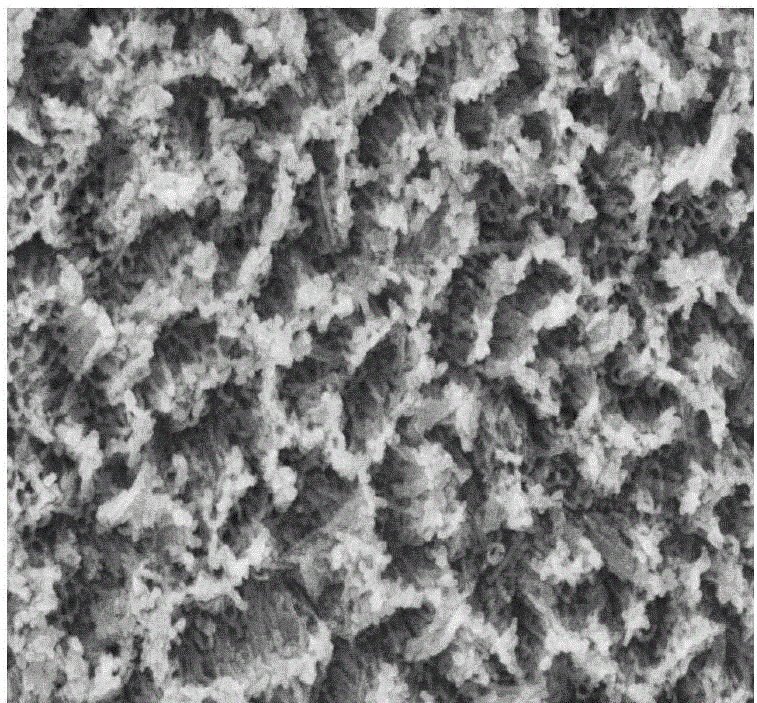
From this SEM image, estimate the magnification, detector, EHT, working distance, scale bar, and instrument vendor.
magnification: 60 K X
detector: SE(M)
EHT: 10 kV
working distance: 9.3 mm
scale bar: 500 nm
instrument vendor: Hitachi